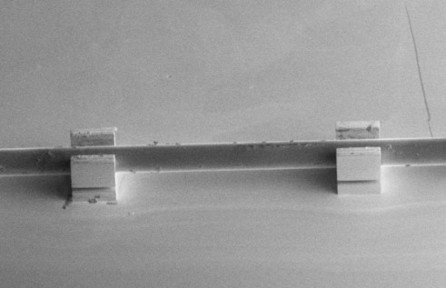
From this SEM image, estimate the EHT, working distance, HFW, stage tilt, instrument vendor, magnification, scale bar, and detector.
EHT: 2 kV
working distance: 4.7 mm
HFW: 2070 µm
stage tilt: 52°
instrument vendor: FEI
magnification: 0.2 K X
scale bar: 500000 nm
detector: ETD